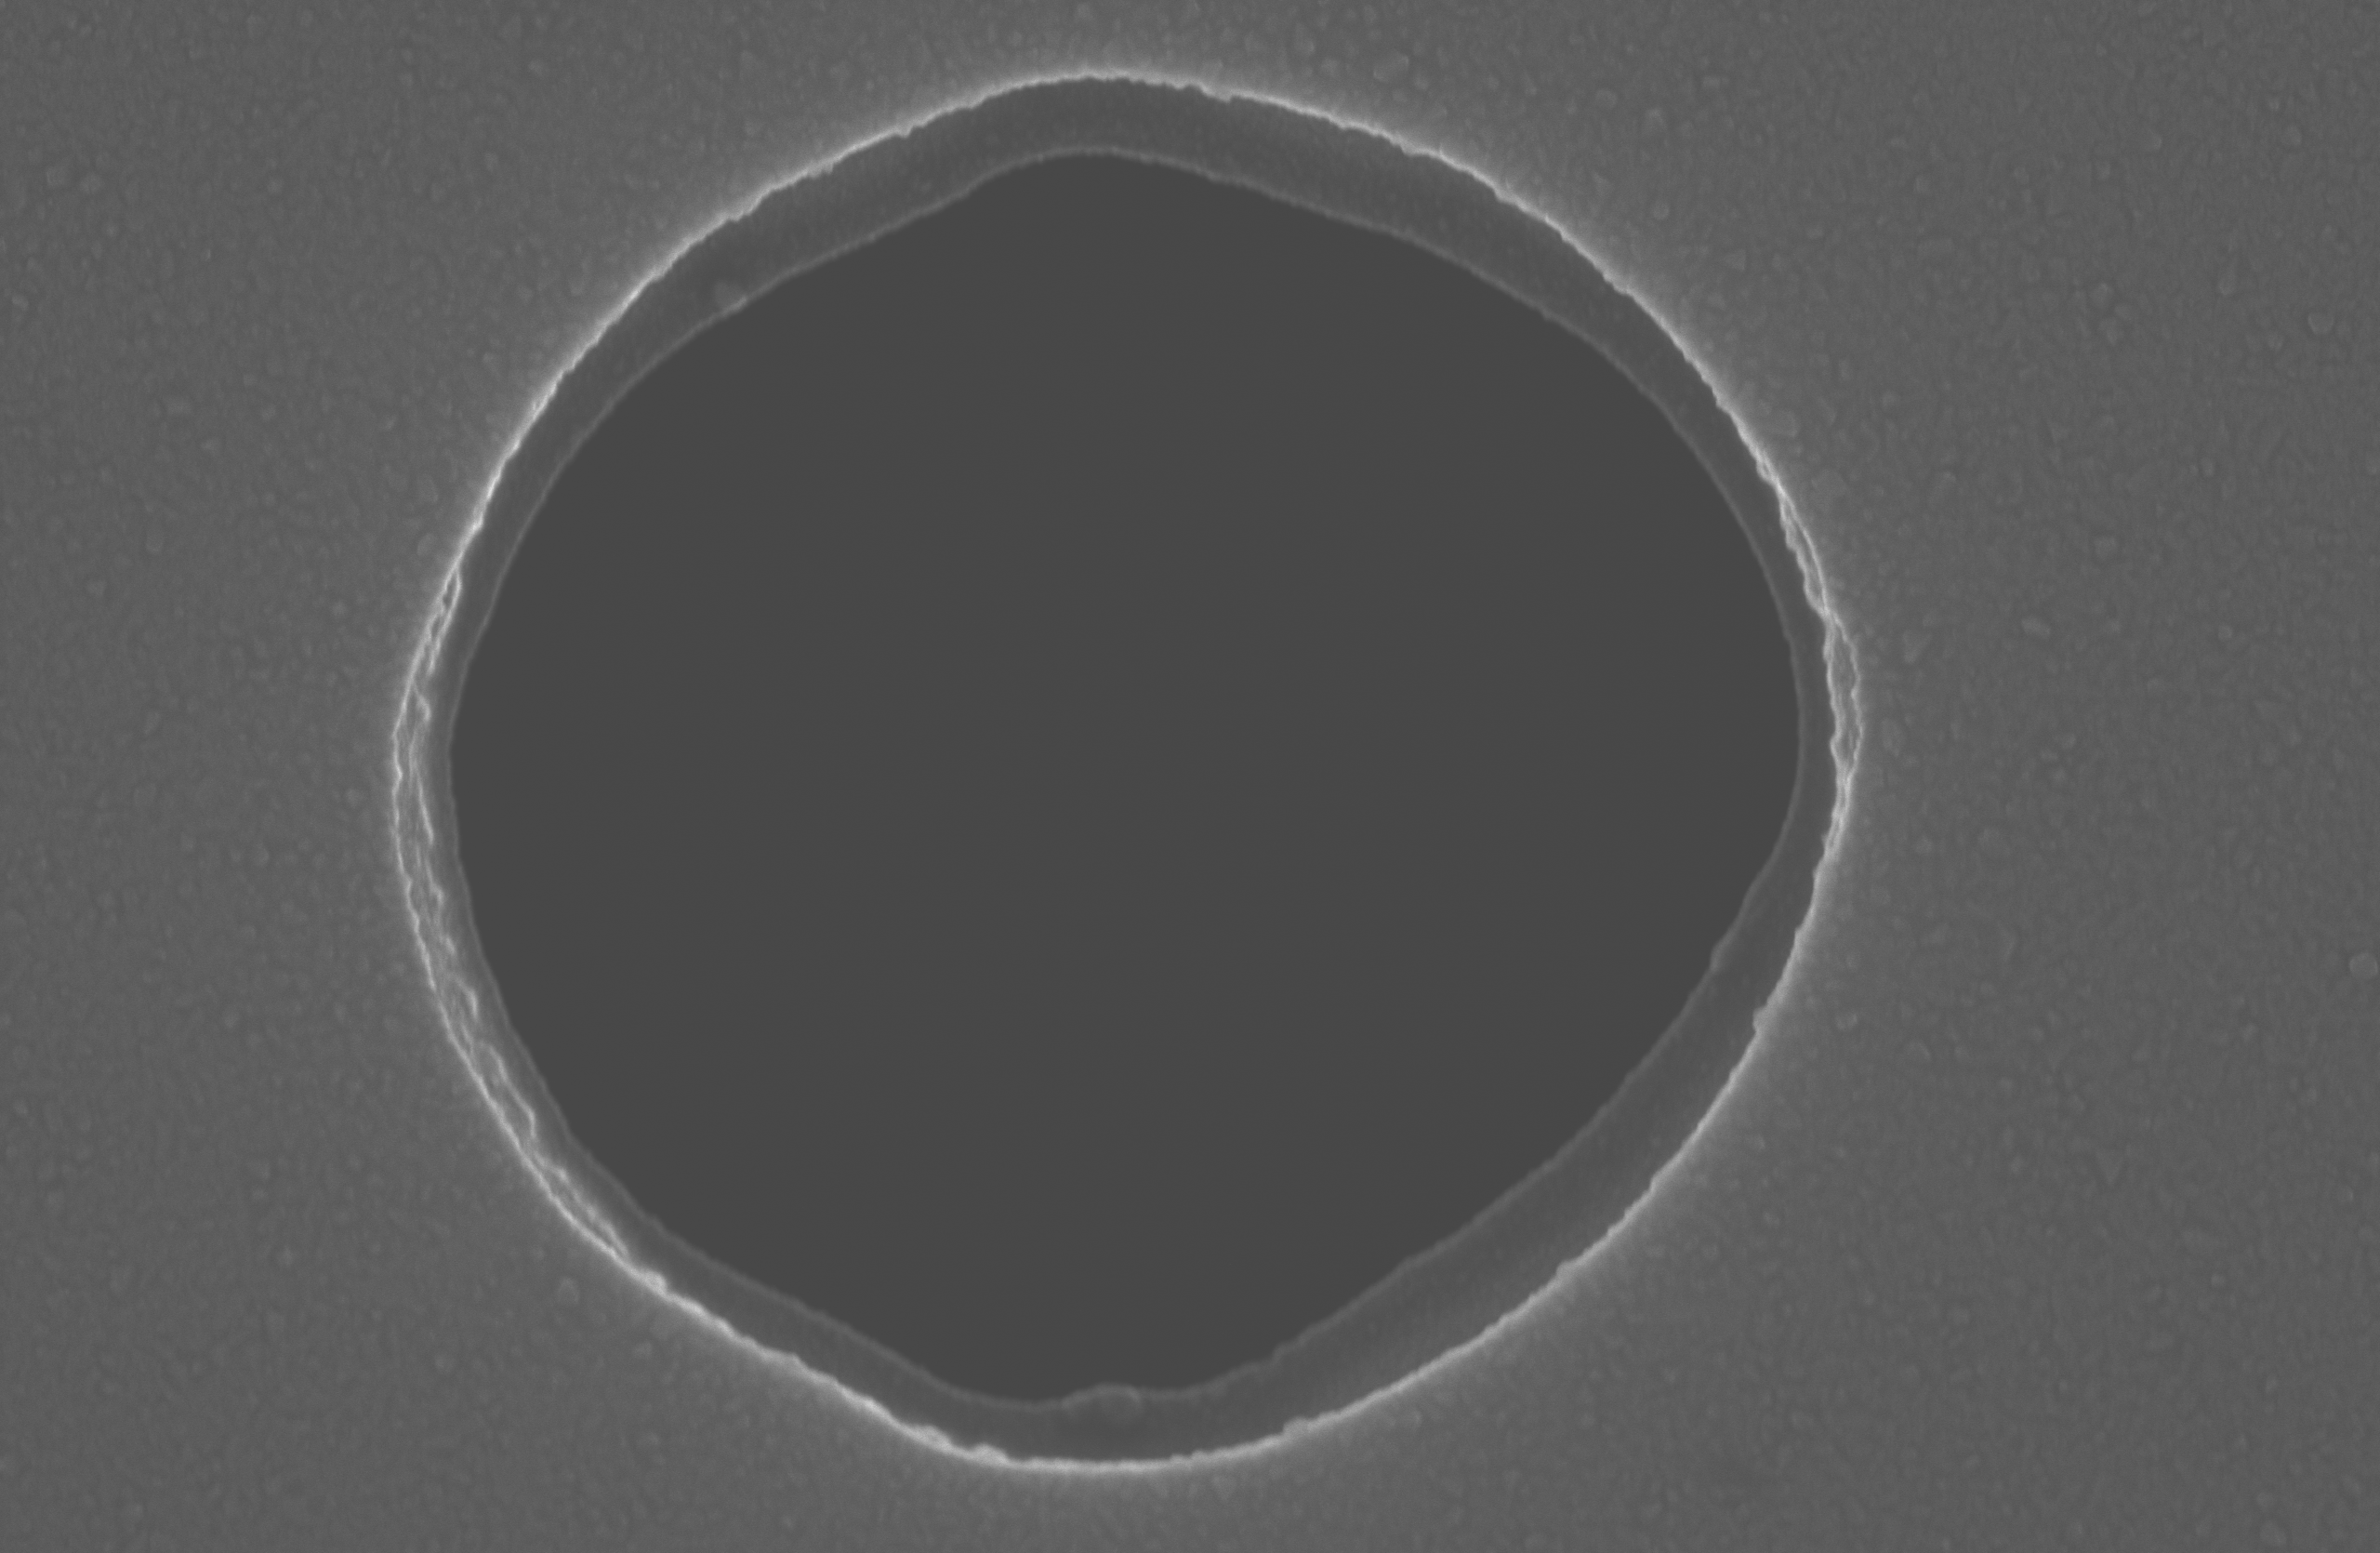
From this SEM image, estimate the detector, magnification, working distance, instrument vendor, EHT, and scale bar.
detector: SE(M)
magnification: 13 K X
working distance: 8.7 mm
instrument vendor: Hitachi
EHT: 20 kV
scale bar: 4000 nm